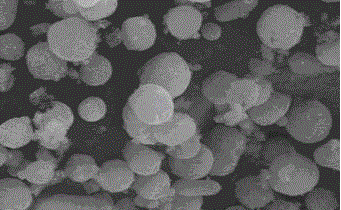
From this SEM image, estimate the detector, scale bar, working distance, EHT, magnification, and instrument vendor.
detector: SE(M)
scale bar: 50000 nm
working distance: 15.6 mm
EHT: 15 kV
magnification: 1 K X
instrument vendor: Hitachi